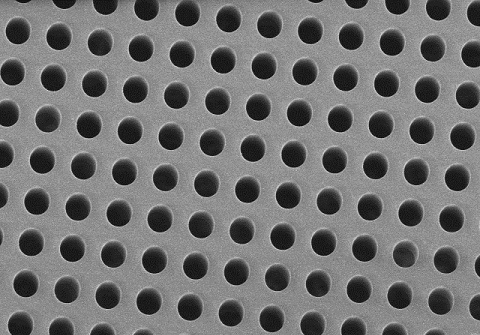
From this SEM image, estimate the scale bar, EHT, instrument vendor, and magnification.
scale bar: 50000 nm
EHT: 3 kV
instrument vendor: Hitachi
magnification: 1 K X